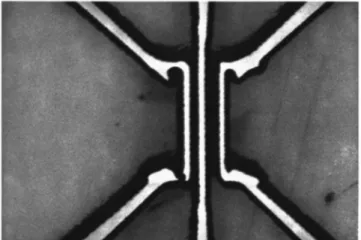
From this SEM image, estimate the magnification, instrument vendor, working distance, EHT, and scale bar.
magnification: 6 K X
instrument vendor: JEOL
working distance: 39 mm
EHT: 15 kV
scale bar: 1000 nm